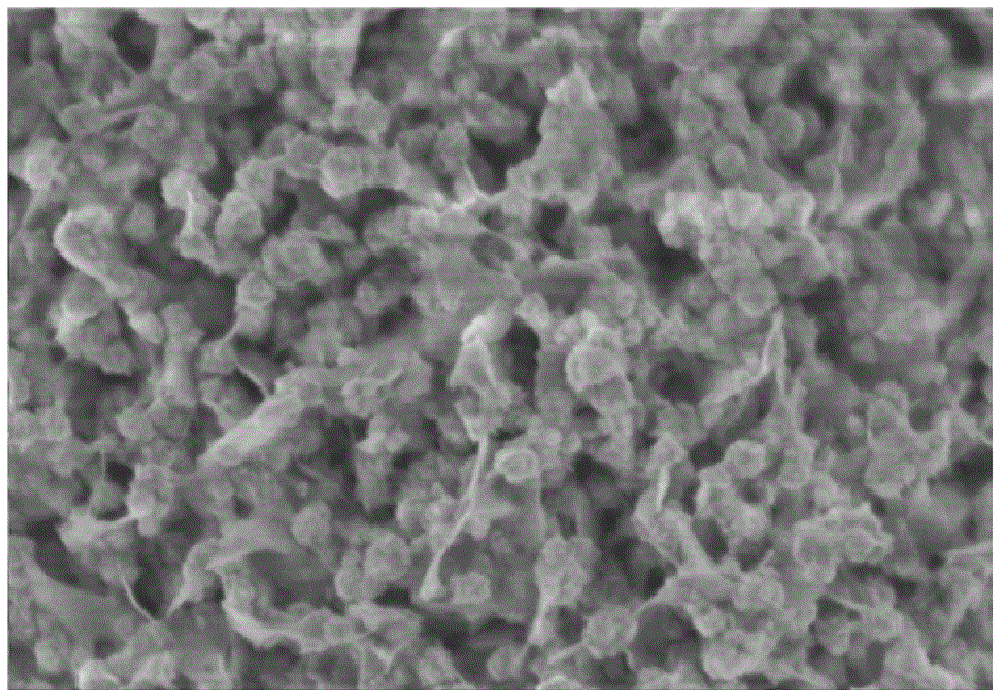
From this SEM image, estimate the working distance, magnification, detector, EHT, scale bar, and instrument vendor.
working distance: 3.7 mm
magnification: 100 K X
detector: SE+BSE(U)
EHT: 1 kV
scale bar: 500 nm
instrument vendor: Hitachi